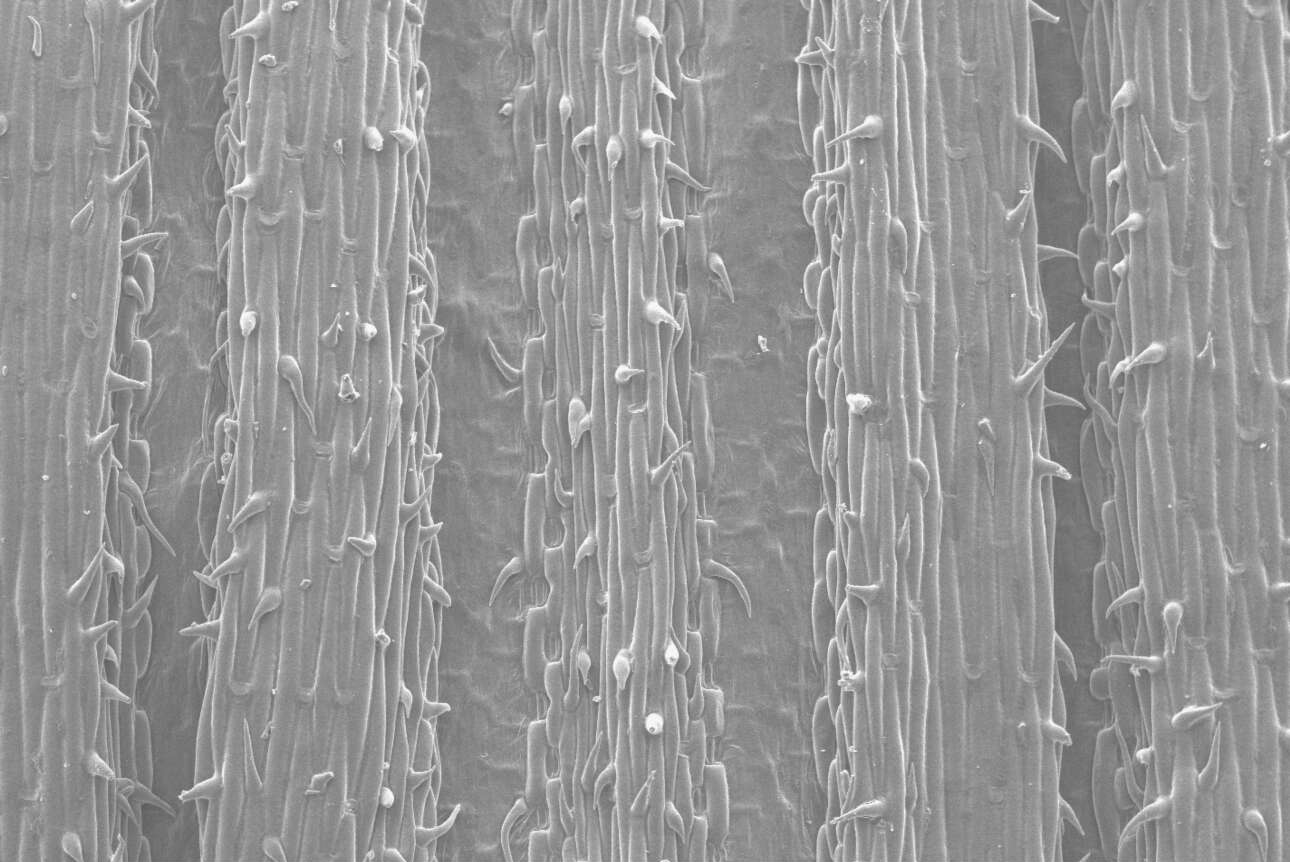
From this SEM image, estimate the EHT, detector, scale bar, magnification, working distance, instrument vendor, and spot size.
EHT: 10 kV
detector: SE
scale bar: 200000 nm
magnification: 0.1 K X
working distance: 9.8 mm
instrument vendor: FEI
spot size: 2